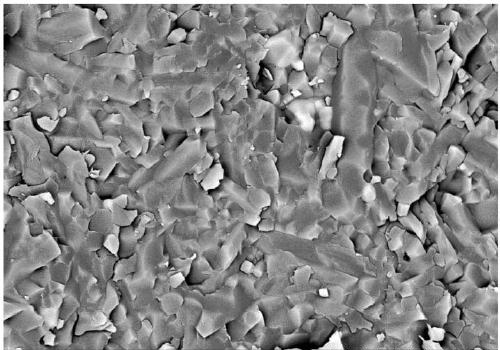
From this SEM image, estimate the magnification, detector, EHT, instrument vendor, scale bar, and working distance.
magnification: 8 K X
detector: PDBSE(3D)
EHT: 10 kV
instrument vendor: Hitachi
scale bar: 5000 nm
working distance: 16.9 mm